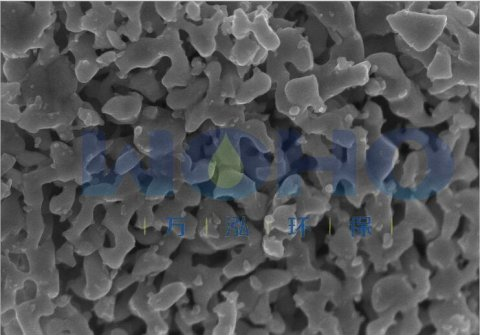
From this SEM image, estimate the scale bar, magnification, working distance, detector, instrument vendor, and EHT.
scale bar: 1000 nm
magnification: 50 K X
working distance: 2.1 mm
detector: SE(U)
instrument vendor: Hitachi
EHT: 5 kV